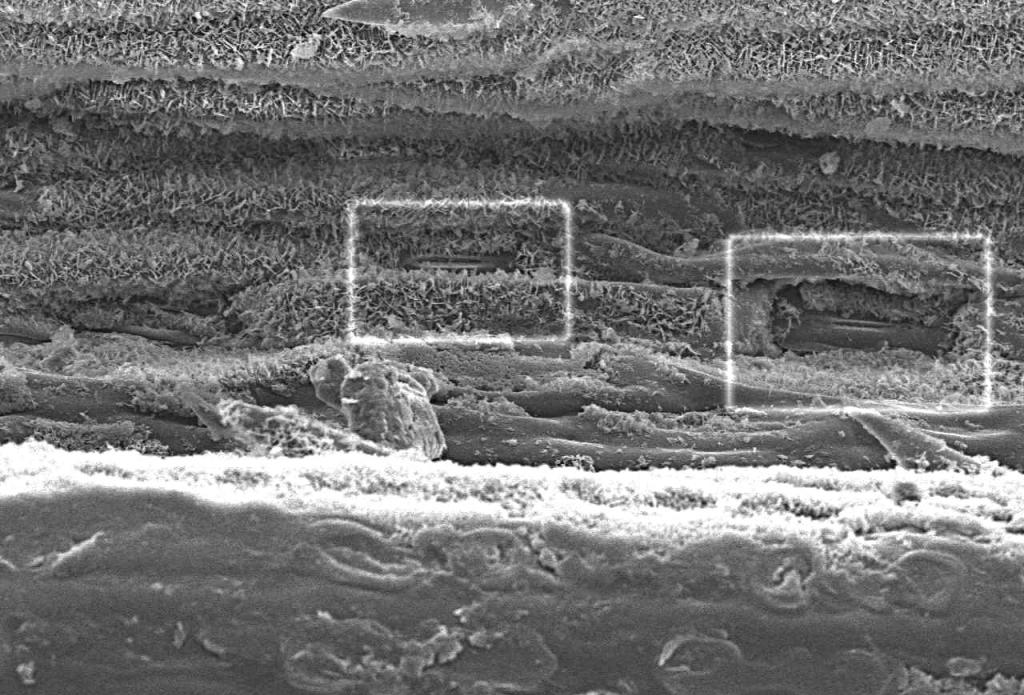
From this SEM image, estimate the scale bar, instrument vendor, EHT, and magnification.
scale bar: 10000 nm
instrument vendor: JEOL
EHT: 9 kV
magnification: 1.2 K X